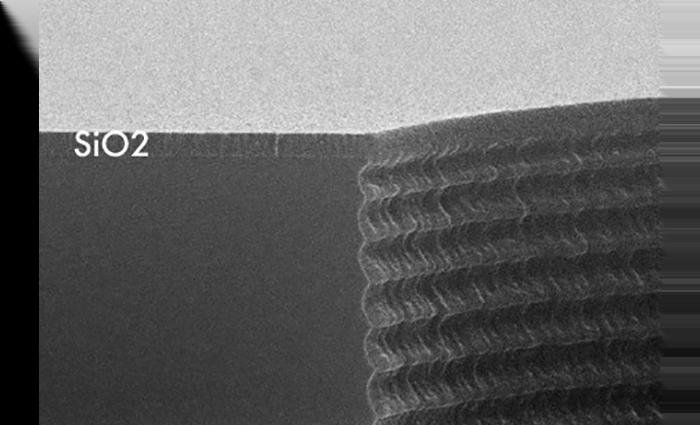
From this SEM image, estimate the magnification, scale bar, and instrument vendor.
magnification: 20 K X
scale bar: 1000 nm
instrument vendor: JEOL